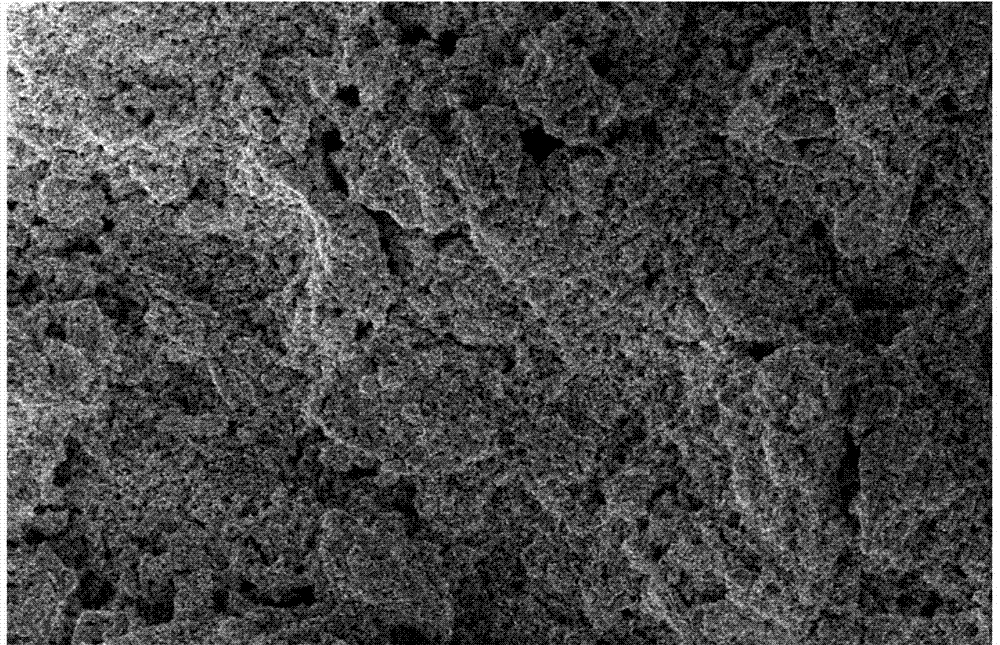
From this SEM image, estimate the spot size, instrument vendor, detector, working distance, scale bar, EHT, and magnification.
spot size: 3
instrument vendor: FEI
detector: SE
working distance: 5 mm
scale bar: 20000 nm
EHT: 5 kV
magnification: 4 K X